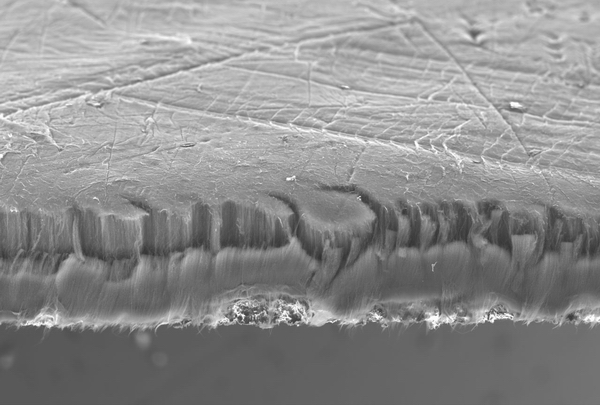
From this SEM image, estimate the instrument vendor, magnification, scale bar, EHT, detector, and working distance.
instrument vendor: JEOL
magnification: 0.1 K X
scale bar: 500000 nm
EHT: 10 kV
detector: SE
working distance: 9.8 mm